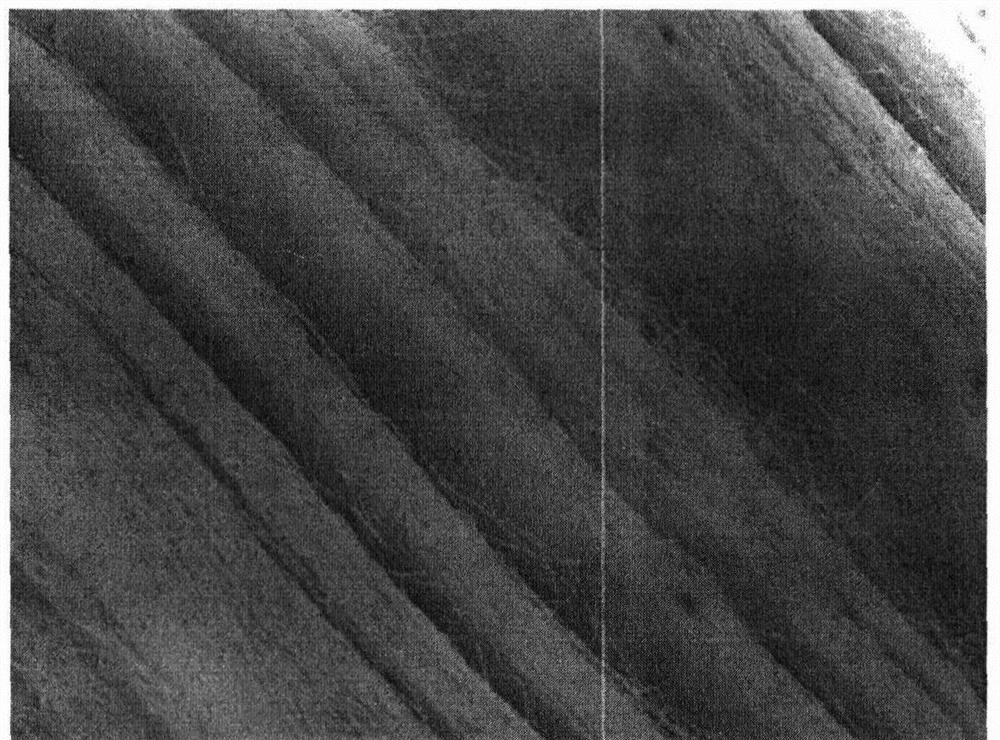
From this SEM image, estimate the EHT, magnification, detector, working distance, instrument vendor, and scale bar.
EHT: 15 kV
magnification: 0.5 K X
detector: LED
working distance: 10.6 mm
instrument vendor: JEOL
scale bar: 10000 nm